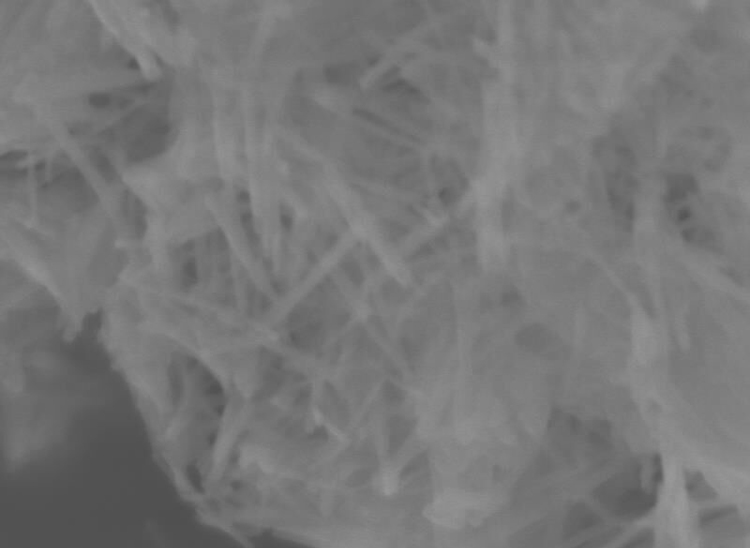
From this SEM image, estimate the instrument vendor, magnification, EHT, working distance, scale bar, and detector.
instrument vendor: JEOL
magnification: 150 K X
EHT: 5 kV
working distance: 3.2 mm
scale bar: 100 nm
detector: SEI